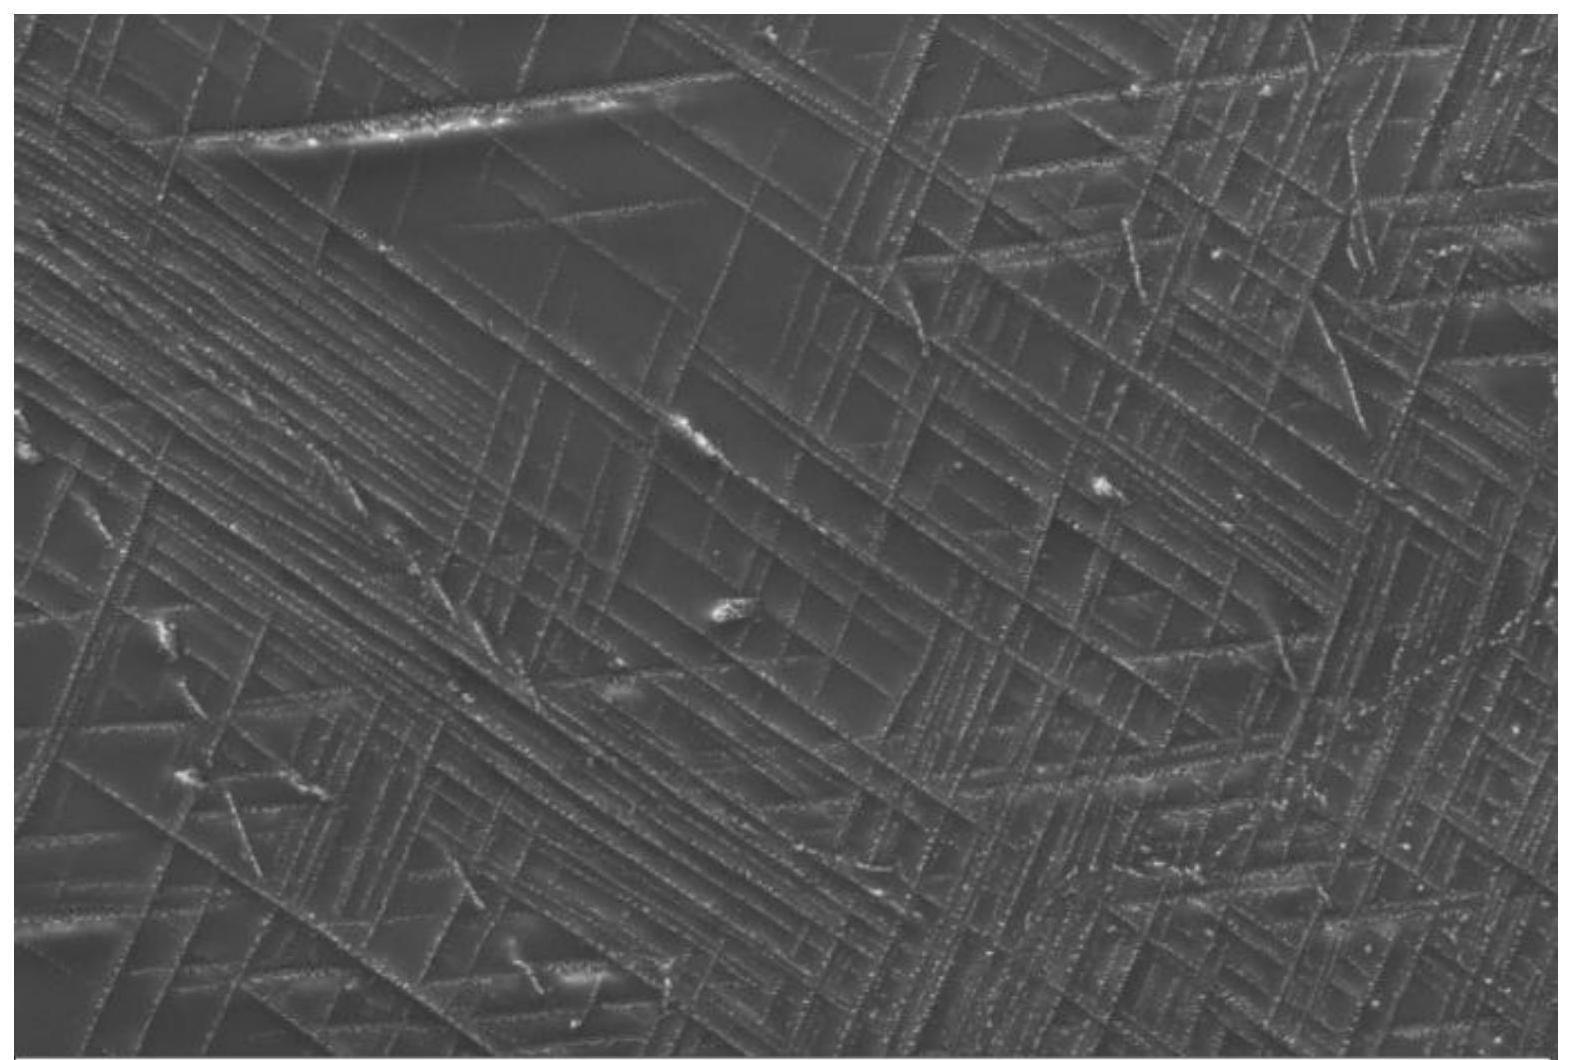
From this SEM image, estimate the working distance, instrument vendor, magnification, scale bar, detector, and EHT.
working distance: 5.5 mm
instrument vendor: Zeiss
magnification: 20 K X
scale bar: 2000 nm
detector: SE2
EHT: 15 kV